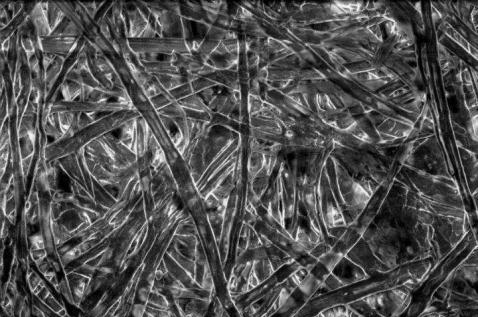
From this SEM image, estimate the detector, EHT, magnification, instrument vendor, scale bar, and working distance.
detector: VPSE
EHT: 15 kV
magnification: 0.5 K X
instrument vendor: Zeiss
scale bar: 20000 nm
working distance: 33 mm